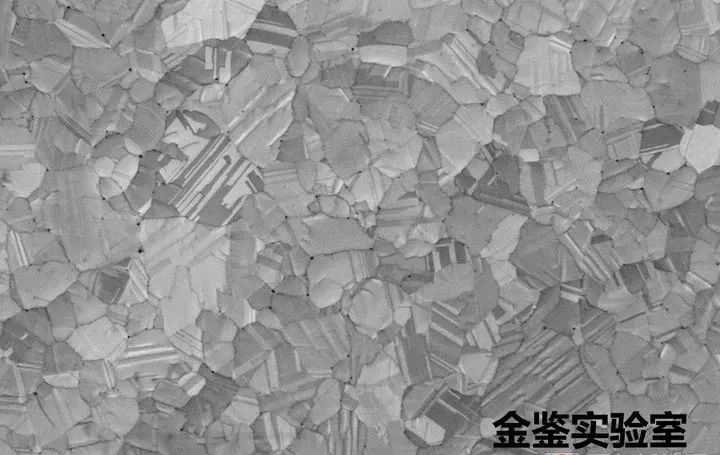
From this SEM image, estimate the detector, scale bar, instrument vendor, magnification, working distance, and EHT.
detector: InLens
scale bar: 1000 nm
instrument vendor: Zeiss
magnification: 10 K X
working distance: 2.9 mm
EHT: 5 kV